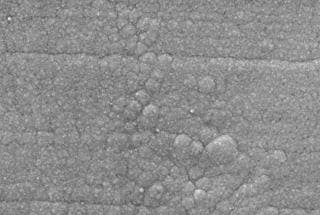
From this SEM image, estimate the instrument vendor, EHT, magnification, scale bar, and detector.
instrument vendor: JEOL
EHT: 15 kV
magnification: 3 K X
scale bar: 5000 nm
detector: SEI